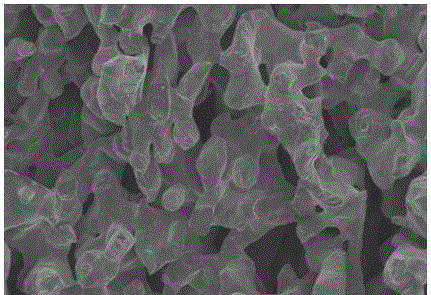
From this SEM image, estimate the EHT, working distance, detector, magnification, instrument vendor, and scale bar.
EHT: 5 kV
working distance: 7 mm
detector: SE(M)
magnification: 0.4 K X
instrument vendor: Hitachi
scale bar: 100000 nm